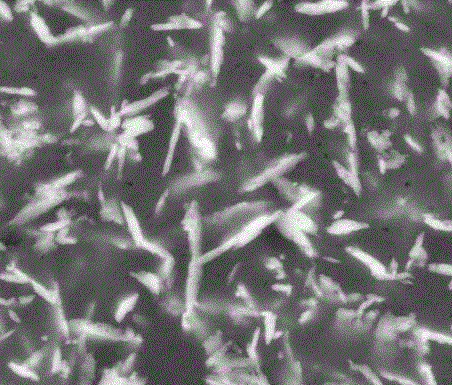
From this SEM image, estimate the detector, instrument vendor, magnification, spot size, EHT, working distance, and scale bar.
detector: BSED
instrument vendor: FEI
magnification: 8 K X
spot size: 5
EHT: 20 kV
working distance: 10.3 mm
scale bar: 5000 nm